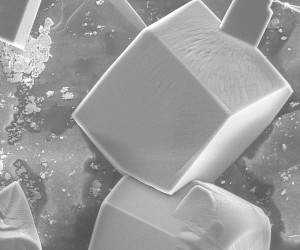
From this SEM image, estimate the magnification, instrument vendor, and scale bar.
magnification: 0.867 K X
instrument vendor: FEI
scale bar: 50000 nm